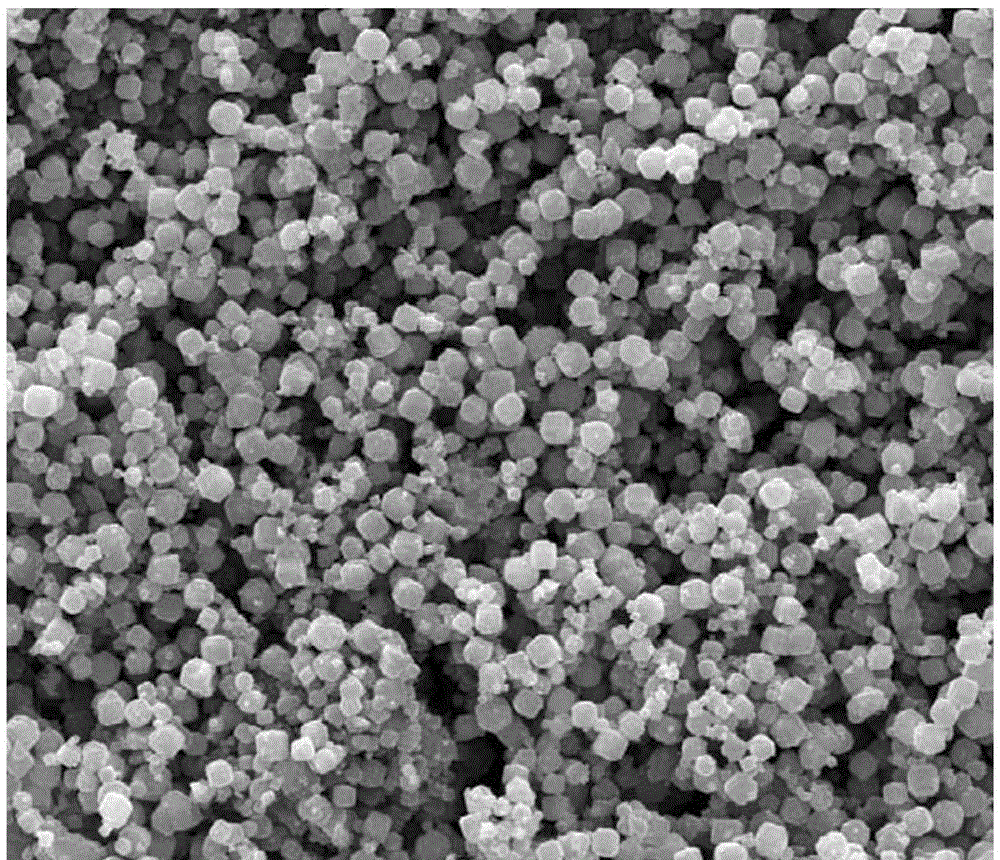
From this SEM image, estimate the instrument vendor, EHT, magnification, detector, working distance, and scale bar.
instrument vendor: FEI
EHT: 30 kV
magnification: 2 K X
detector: SE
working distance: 9.3 mm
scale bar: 50000 nm